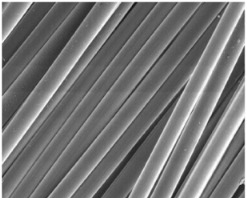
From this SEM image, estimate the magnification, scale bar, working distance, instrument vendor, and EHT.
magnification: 1 K X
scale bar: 100000 nm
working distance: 10.1 mm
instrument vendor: FEI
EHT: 10 kV